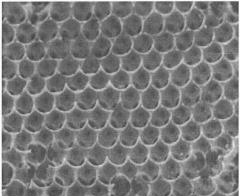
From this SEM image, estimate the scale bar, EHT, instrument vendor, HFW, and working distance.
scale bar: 3000 nm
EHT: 30 kV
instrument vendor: FEI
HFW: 7.46 µm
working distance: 13.8 mm